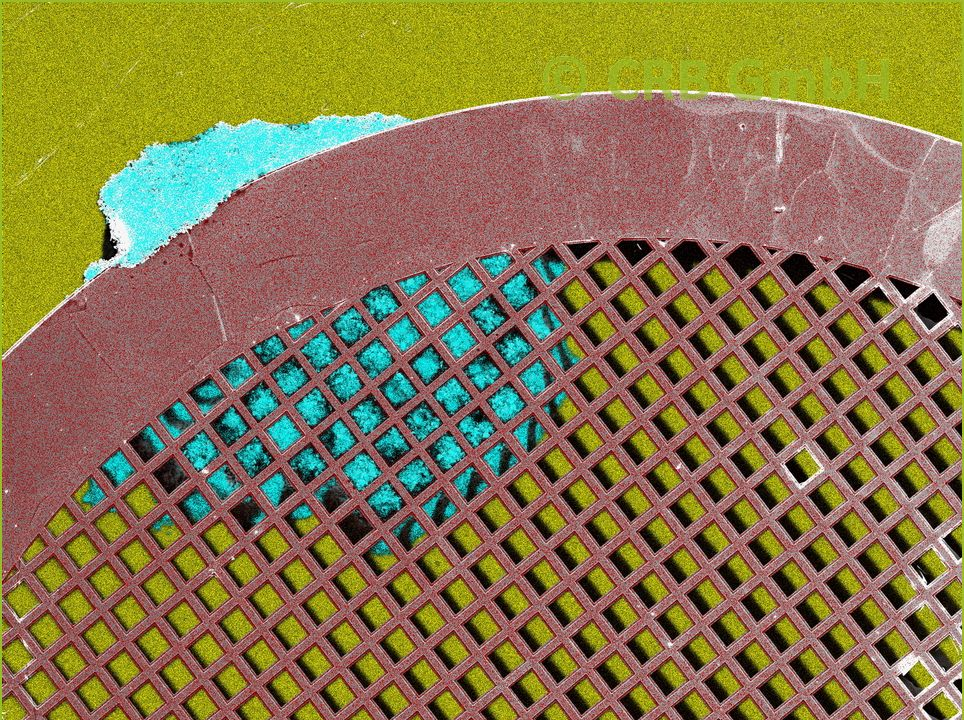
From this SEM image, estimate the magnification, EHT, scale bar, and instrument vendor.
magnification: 0.062 K X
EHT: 20 kV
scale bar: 200000 nm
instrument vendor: other